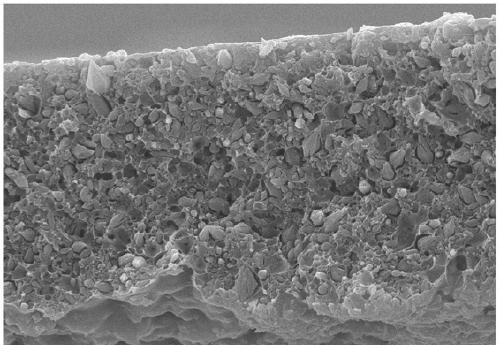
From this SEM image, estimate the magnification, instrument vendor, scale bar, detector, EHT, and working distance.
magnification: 2 K X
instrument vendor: Hitachi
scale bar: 20000 nm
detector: SE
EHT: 20 kV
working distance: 10.1 mm